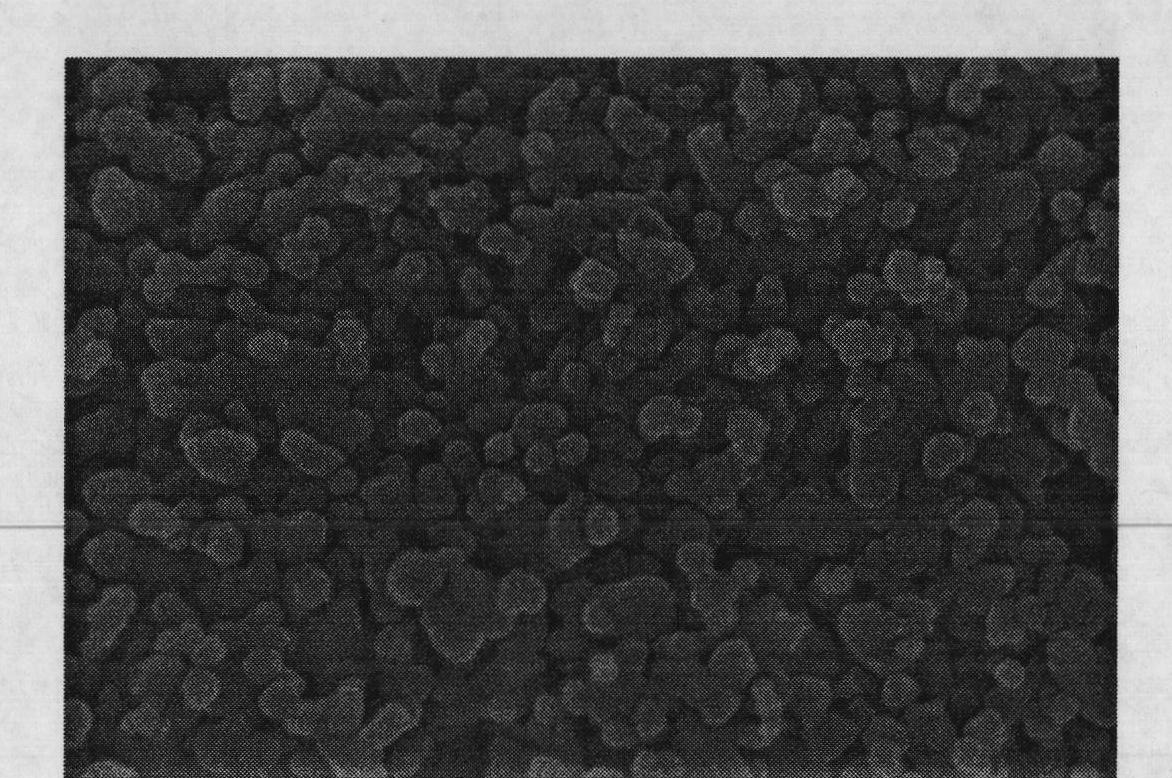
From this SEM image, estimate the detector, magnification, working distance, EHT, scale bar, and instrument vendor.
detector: SE(U)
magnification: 100 K X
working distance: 4 mm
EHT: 8 kV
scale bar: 500 nm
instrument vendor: Hitachi